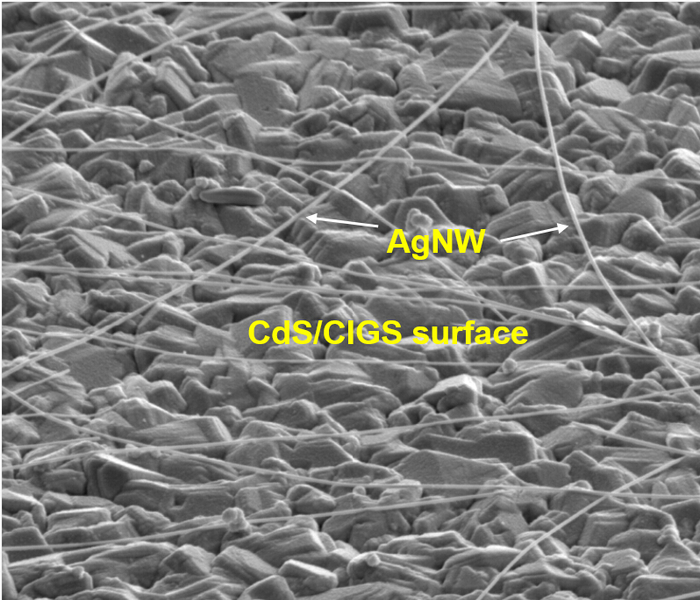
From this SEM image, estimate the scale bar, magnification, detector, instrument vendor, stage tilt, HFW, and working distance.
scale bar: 5000 nm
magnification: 20 K X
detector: ETD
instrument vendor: FEI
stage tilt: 60°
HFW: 12.8 µm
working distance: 4.9 mm